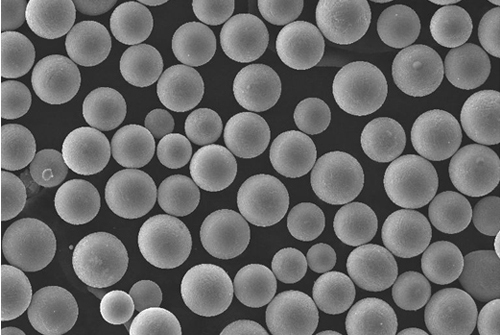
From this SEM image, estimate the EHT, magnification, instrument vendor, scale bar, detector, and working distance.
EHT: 30 kV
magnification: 0.5 K X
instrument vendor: Tescan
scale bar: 100000 nm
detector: SE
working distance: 5.46 mm